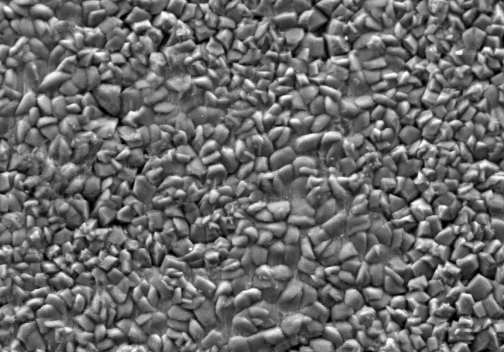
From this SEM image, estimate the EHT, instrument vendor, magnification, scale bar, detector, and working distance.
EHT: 15 kV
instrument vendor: Hitachi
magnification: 1 K X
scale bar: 50000 nm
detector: SE M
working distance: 10.6 mm